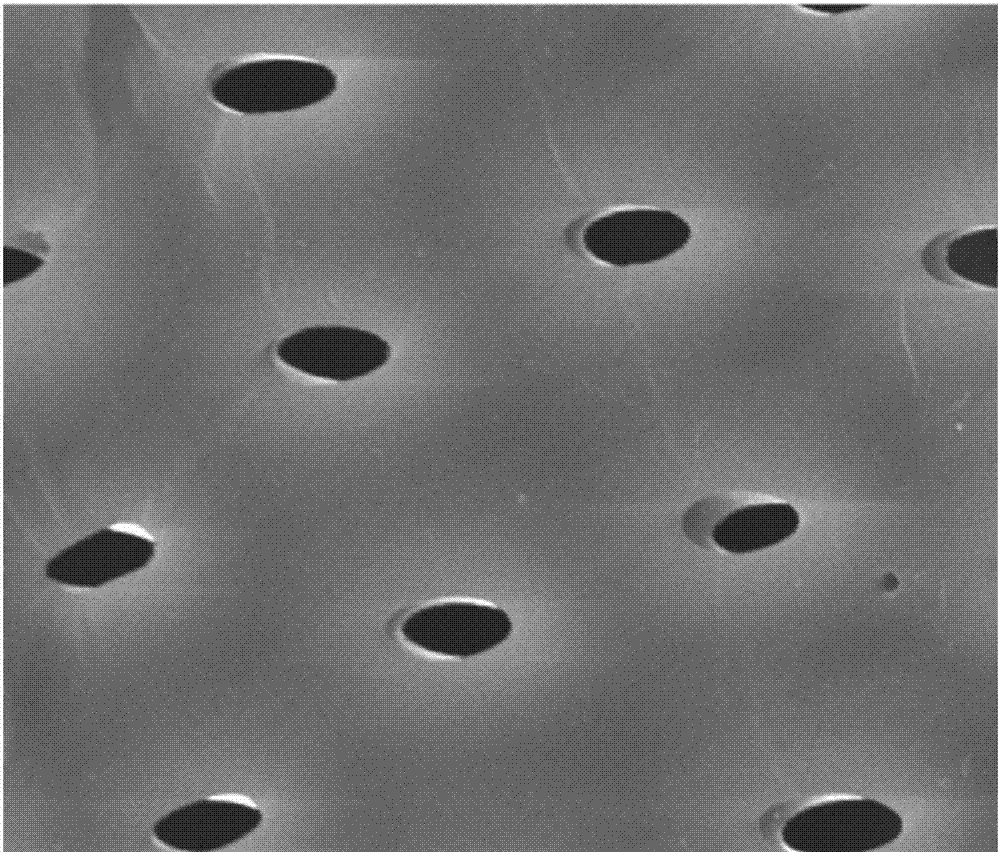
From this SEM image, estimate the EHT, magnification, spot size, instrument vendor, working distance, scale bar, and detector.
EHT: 12.5 kV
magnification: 5 K X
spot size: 3.5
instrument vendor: FEI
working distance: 9.6 mm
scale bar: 5000 nm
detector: ETD